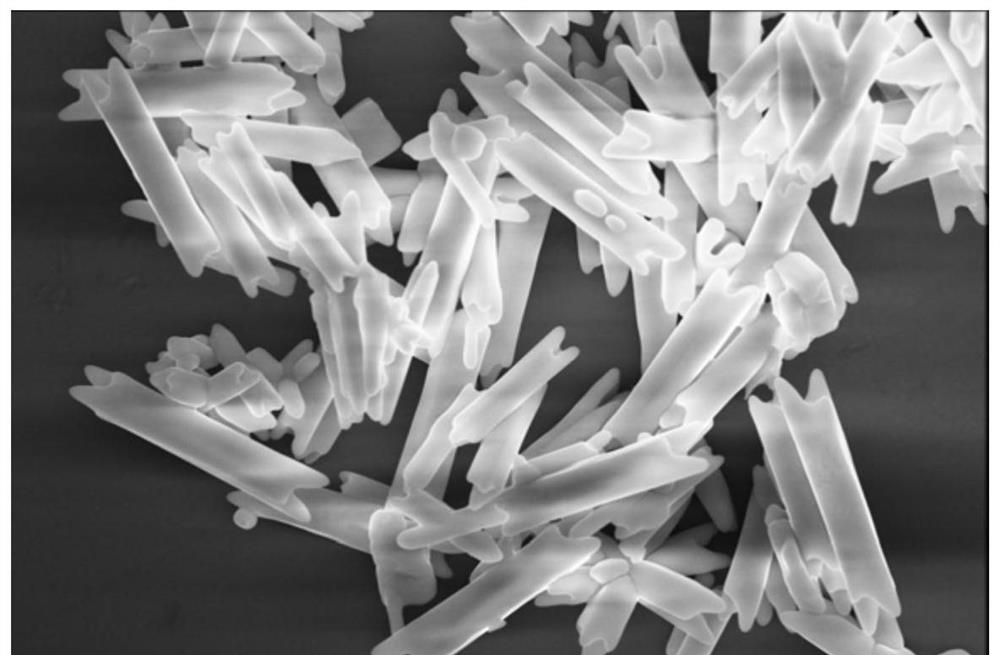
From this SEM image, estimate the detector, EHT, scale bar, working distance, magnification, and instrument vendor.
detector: TLD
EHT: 10 kV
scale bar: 10000 nm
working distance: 3.5 mm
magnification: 12 K X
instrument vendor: FEI